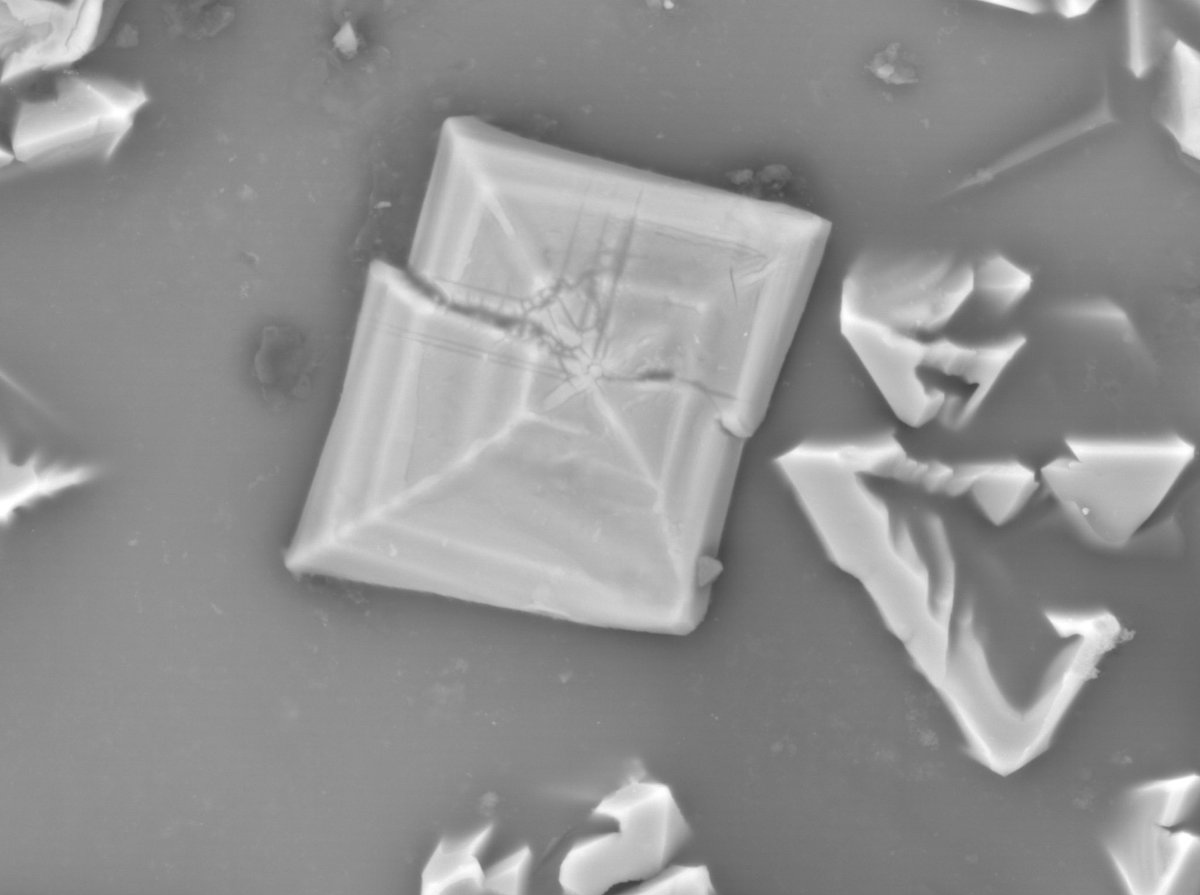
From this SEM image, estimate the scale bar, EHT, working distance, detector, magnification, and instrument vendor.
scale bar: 1000 nm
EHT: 15 kV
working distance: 11 mm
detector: COMPO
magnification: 3.5 K X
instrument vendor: JEOL